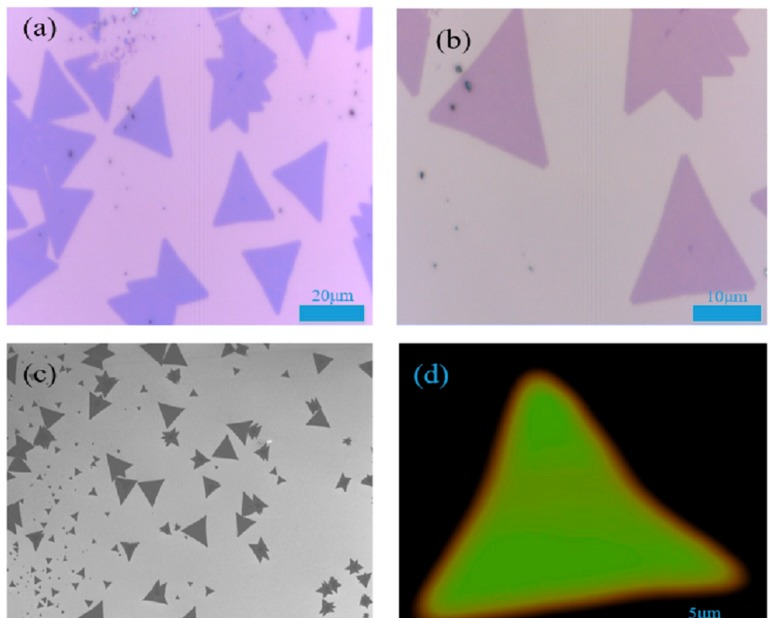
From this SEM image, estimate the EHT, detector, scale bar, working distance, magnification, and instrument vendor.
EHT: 5 kV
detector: SEI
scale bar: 10000 nm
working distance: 8.1 mm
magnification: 0.5 K X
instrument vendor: JEOL